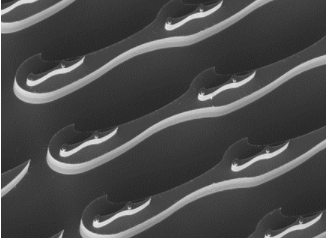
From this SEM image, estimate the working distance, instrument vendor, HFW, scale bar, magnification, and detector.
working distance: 19.9 mm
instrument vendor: Tescan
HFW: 519 µm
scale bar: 100000 nm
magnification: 0.4 K X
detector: SE